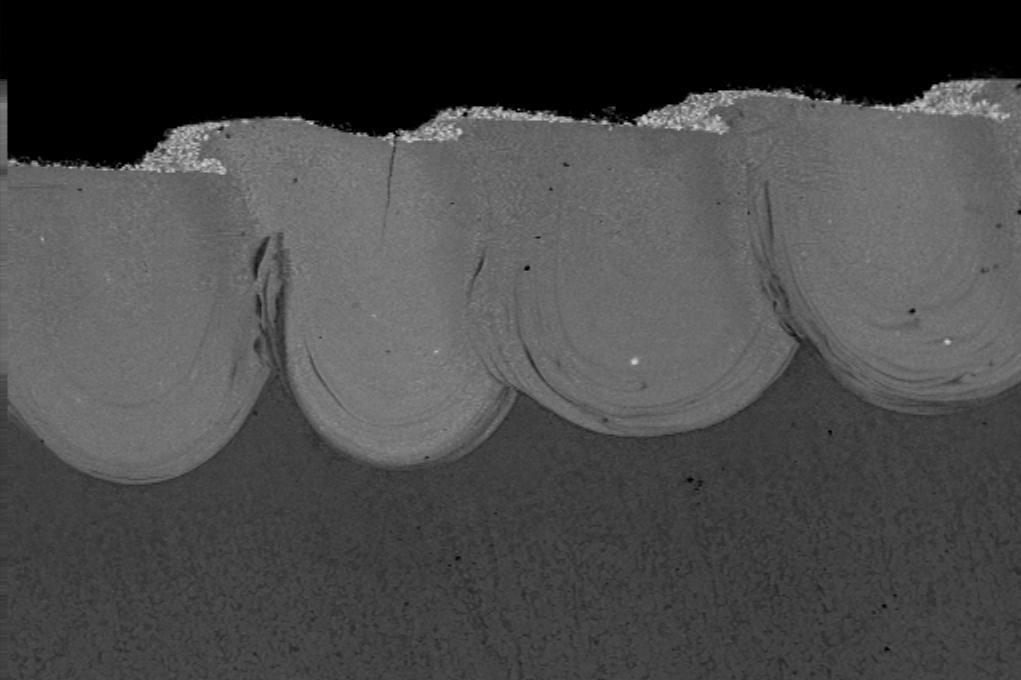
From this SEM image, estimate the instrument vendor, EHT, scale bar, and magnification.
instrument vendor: other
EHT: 23 kV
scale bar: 1000000 nm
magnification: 0.025 K X